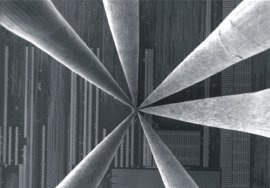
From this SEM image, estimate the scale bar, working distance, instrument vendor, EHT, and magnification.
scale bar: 30000 nm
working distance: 10 mm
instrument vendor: Zeiss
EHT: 0.8 kV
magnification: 0.6 K X